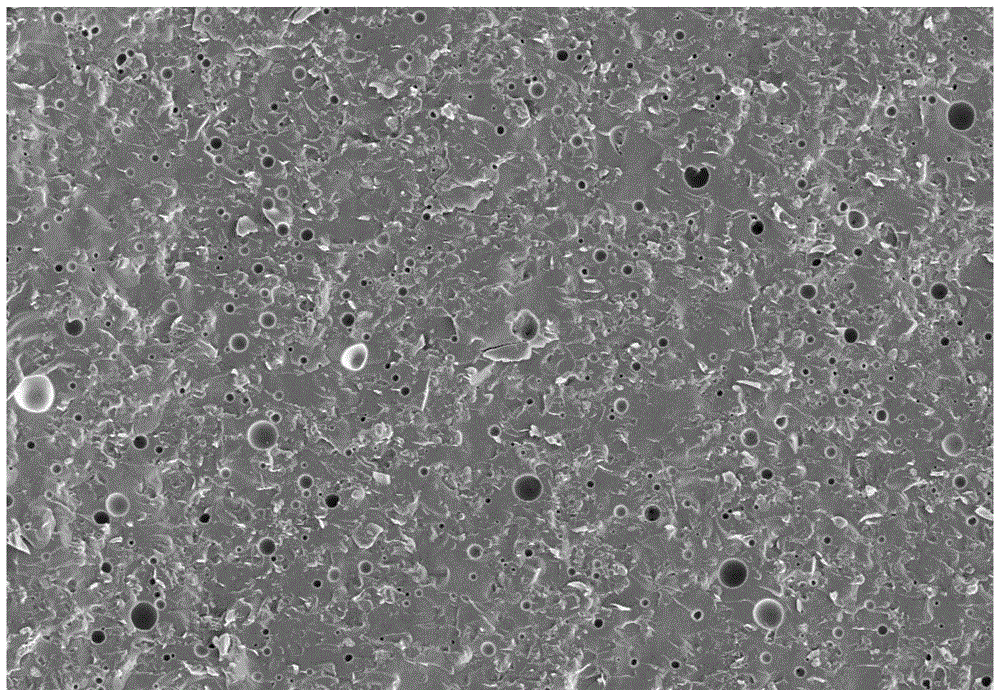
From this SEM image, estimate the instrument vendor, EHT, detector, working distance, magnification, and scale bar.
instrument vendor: Zeiss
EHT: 5 kV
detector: SE2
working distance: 15.2 mm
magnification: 1 K X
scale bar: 3000 nm